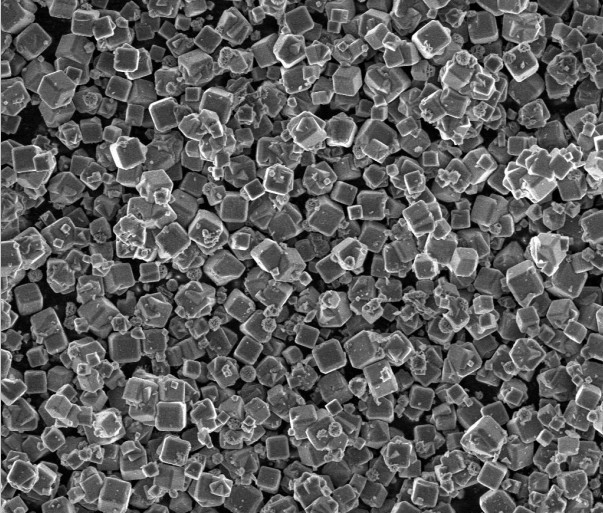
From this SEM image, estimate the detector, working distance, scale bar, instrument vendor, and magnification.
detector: TLD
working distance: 4.9 mm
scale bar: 30000 nm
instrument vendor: FEI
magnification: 2 K X